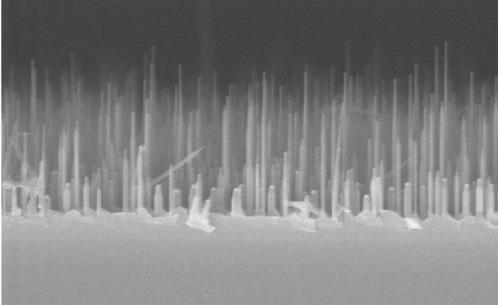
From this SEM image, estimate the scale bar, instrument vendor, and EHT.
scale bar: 1000 nm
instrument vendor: JEOL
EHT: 20 kV